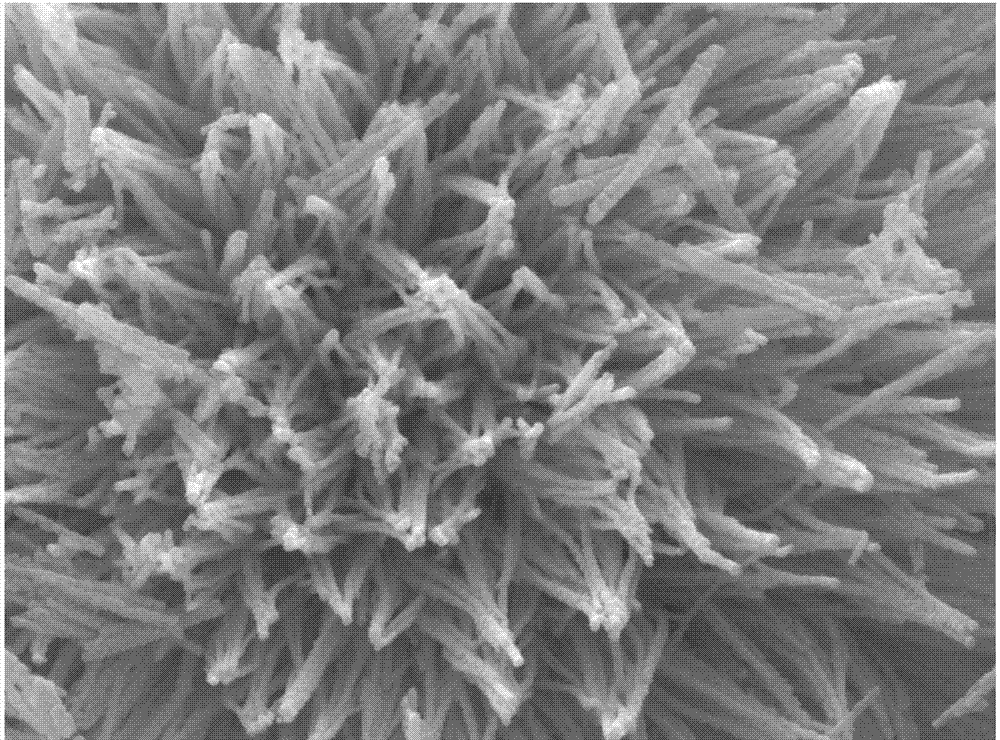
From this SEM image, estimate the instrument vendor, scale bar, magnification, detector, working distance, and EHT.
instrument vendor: JEOL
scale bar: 100 nm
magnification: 50 K X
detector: SEI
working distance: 5.9 mm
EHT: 15 kV